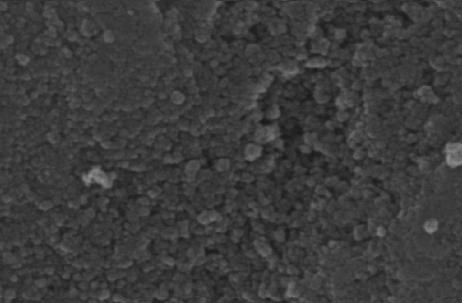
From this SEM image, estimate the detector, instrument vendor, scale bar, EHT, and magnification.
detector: SE1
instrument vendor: Zeiss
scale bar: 1000 nm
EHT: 20 kV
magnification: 30 K X